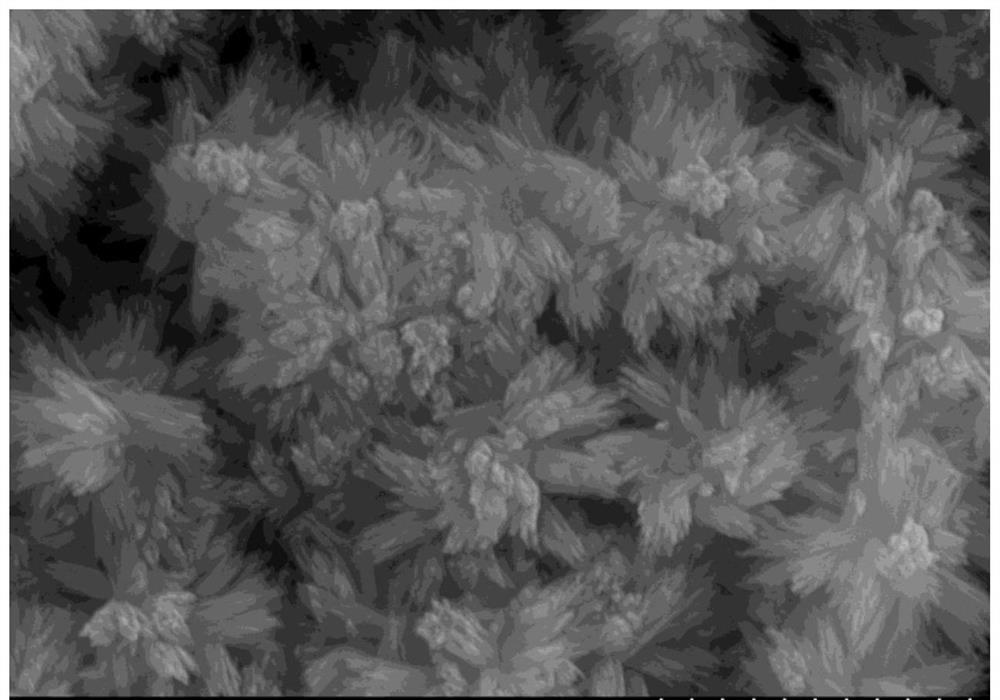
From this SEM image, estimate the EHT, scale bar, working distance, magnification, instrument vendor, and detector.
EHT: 20 kV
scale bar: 2000 nm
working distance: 11 mm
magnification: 20 K X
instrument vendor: Hitachi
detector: SE